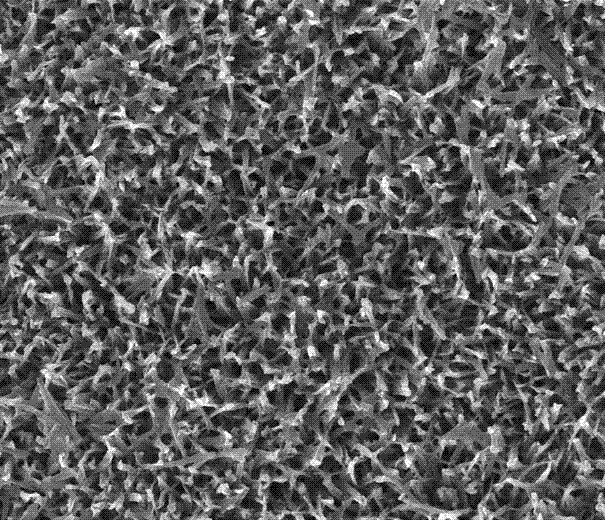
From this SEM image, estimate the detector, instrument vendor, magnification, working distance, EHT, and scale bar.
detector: TLD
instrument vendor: FEI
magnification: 50 K X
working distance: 5.1 mm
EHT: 10 kV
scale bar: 2000 nm